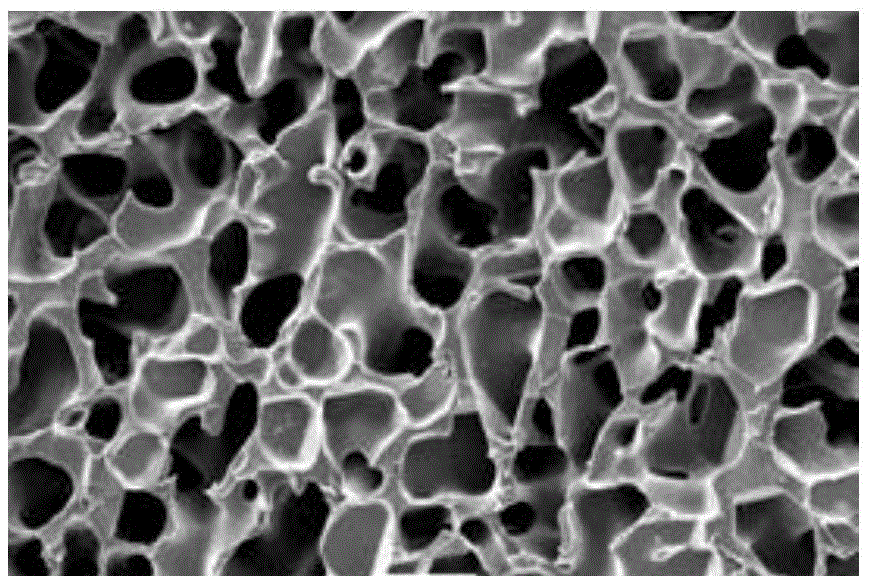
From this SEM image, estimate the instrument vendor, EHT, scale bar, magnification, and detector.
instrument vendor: JEOL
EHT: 10 kV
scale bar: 50000 nm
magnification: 0.3 K X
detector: SEI